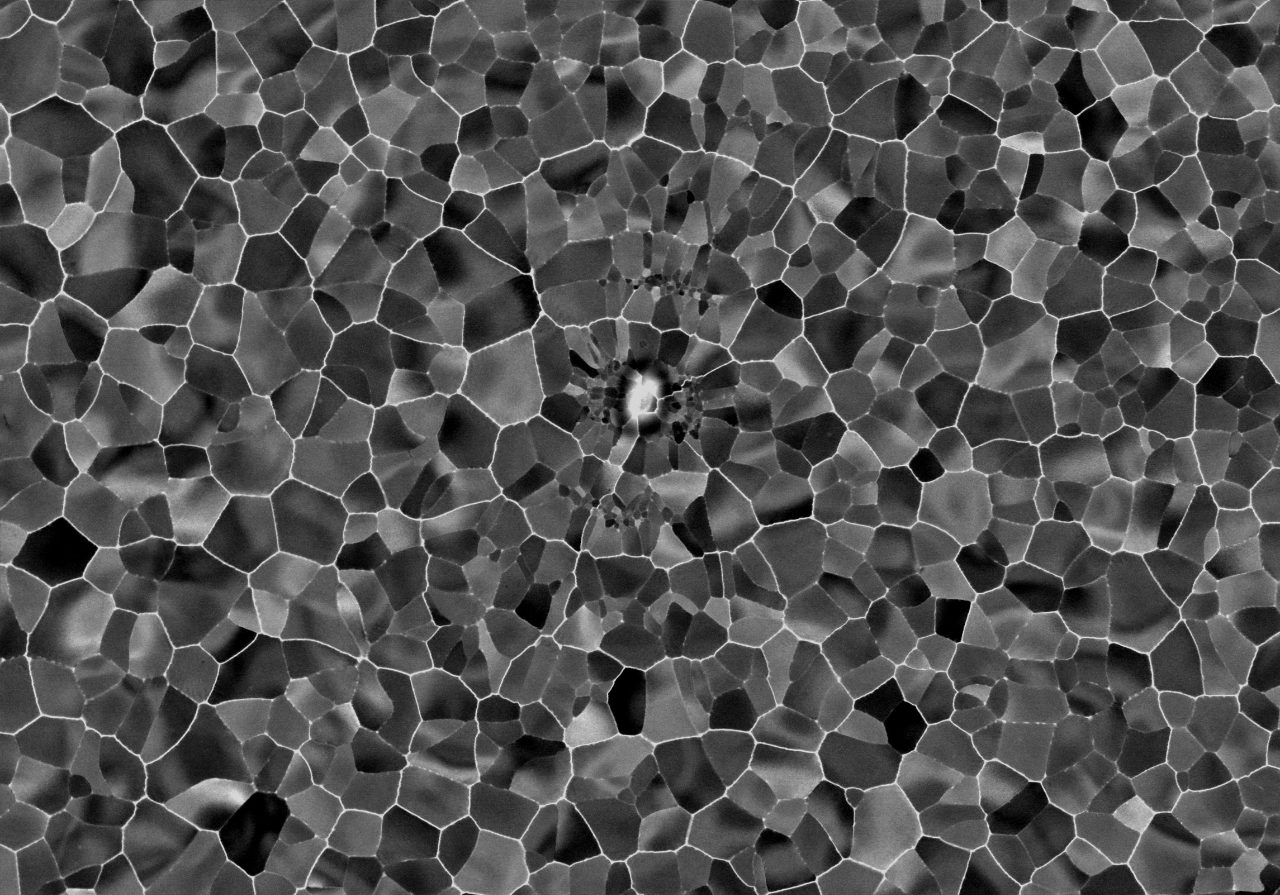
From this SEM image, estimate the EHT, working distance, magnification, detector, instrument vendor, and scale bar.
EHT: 30 kV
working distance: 3.7 mm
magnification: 10 K X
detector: Argus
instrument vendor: other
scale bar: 2000 nm